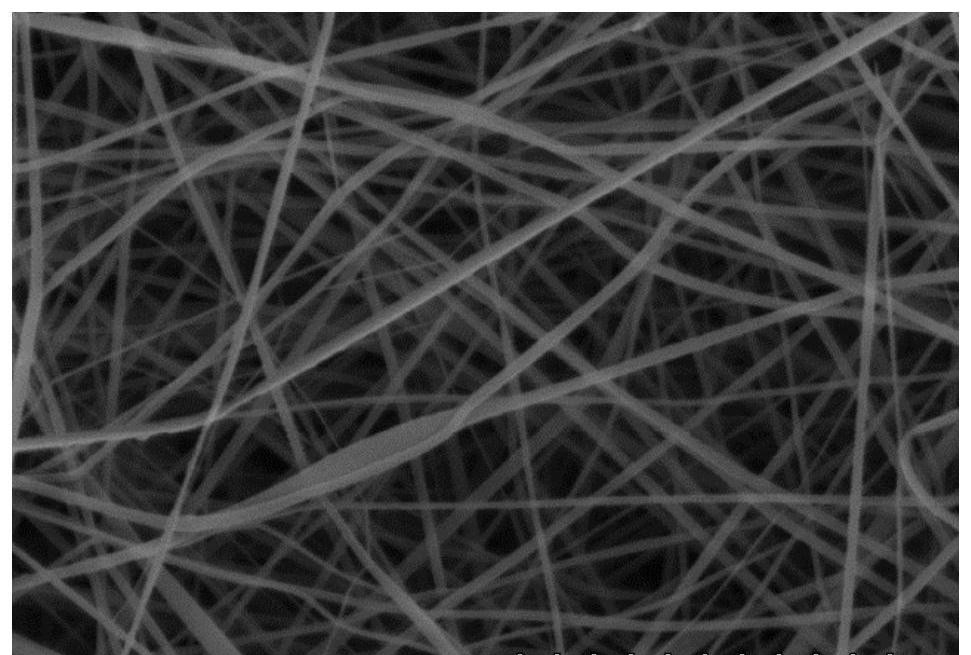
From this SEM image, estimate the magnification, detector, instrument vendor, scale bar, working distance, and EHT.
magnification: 5 K X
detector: SE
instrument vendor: Hitachi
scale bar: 10000 nm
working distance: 18.3 mm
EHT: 15 kV